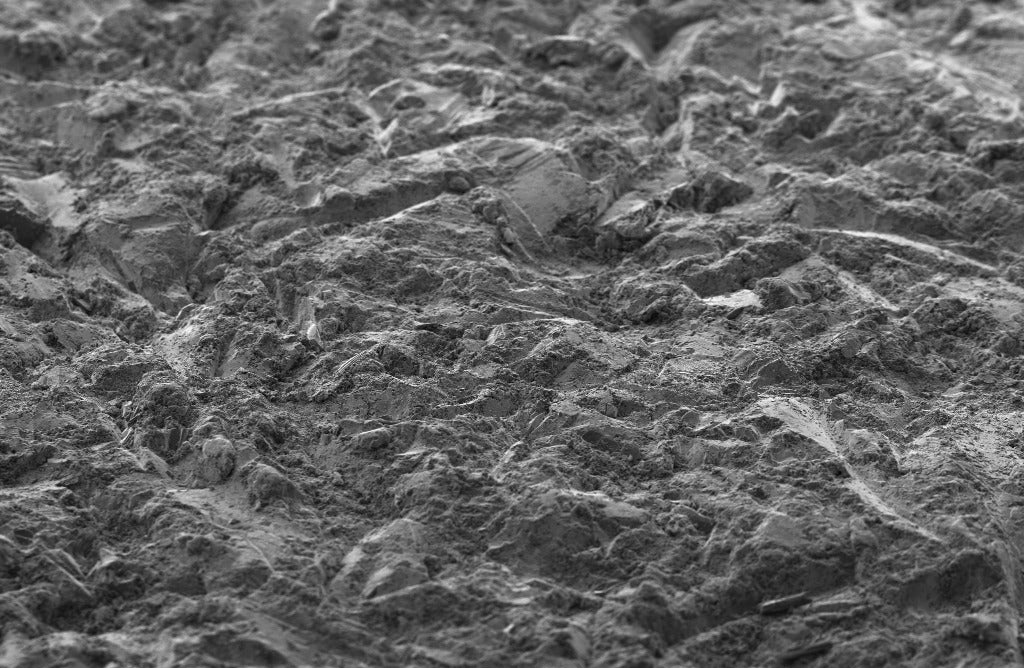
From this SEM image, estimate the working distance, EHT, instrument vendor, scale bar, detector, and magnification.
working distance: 6.6 mm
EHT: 5 kV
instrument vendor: Zeiss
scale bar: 20000 nm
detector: SE2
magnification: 0.2 K X